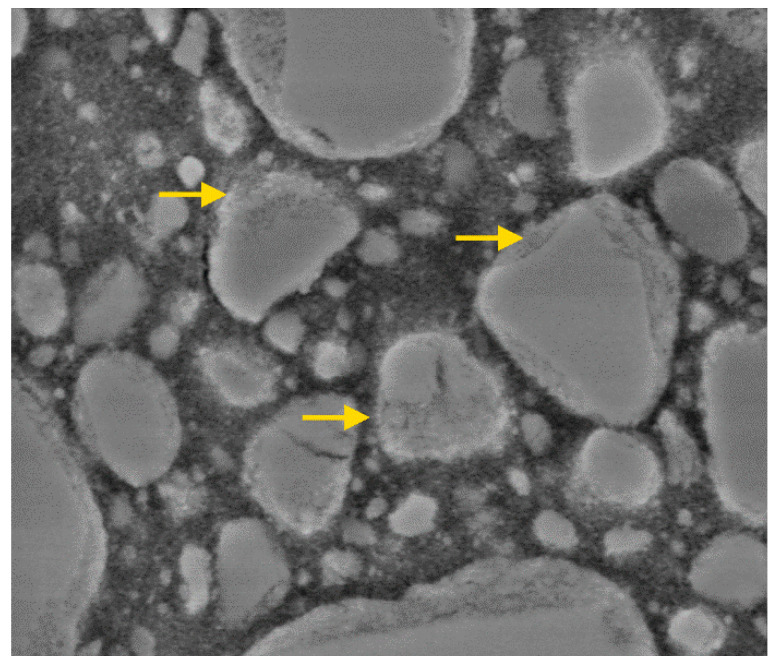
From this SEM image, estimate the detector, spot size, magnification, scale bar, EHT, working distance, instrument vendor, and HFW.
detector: BSE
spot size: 3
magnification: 50 K X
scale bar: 1000 nm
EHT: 8 kV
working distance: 9.4 mm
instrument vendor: FEI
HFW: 5.41 µm